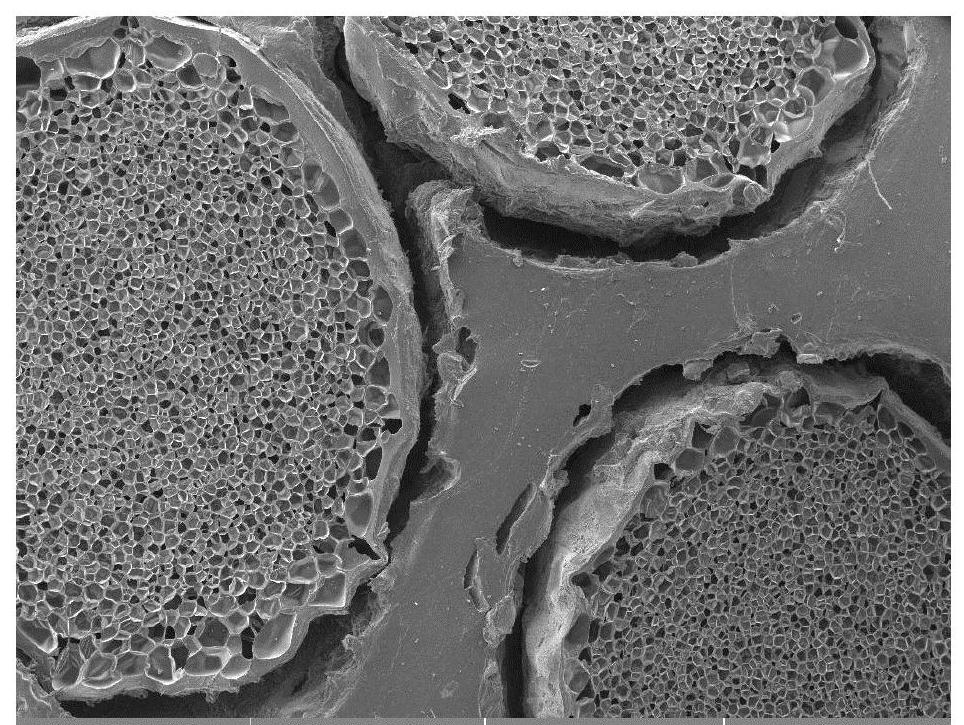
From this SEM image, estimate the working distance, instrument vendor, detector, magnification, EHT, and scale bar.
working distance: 13.72 mm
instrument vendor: Tescan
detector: SE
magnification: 0.035 K X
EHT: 12 kV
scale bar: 2000000 nm